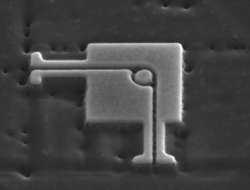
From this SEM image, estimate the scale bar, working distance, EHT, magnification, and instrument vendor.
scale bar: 1000 nm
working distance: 4.2 mm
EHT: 15 kV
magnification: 50 K X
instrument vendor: FEI